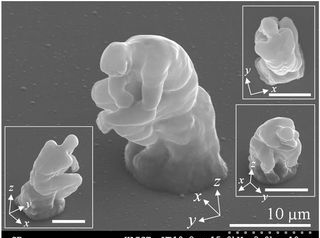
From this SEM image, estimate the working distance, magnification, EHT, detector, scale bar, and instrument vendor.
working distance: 10.8 mm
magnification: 3 K X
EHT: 15 kV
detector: SE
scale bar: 10000 nm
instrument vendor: Hitachi